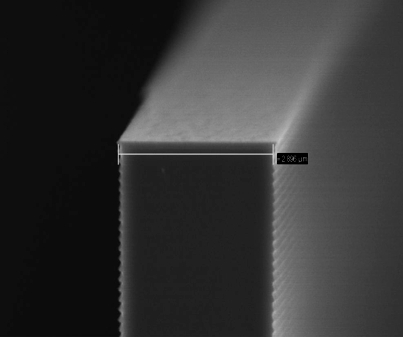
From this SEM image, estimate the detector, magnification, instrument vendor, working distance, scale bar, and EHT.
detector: InLens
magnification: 43.46 K X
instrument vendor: Zeiss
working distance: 3.7 mm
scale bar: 200 nm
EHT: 5 kV